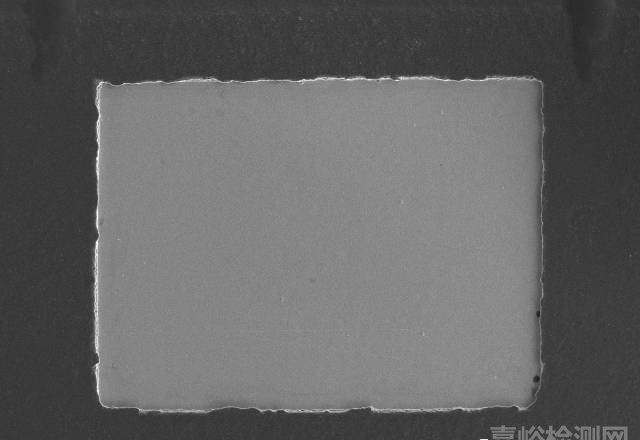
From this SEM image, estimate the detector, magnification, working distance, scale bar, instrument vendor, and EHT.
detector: SE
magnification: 0.05 K X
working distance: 10 mm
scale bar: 1000000 nm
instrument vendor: Hitachi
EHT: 15 kV